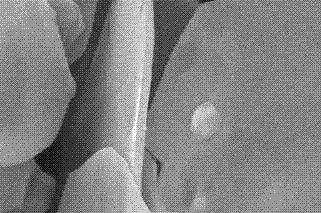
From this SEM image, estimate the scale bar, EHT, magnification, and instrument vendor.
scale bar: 1000 nm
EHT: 20 kV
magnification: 10 K X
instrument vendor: JEOL